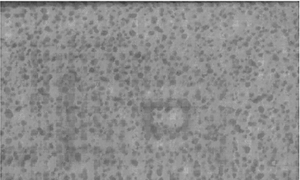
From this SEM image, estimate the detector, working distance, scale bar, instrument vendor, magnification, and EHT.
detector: SE(U)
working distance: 2.9 mm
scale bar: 500 nm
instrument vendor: Hitachi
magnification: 100 K X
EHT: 1.5 kV